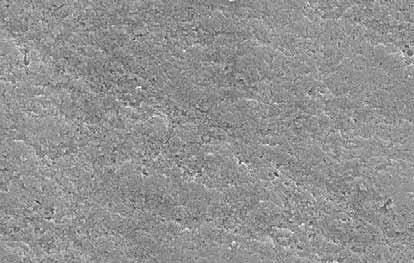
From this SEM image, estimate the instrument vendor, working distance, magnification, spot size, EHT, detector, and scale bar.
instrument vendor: FEI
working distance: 9.6 mm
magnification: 20 K X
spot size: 3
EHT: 25 kV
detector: SE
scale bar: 1000 nm